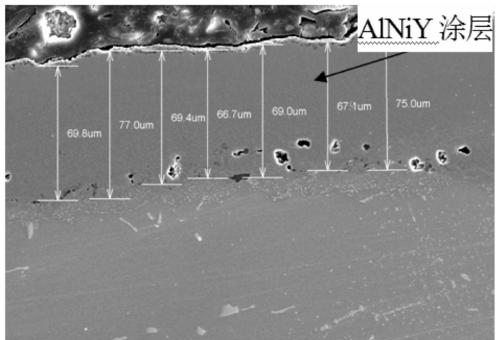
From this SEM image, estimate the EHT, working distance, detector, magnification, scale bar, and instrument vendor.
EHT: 20 kV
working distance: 11.5 mm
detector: SE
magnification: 0.5 K X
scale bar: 100000 nm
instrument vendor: Hitachi